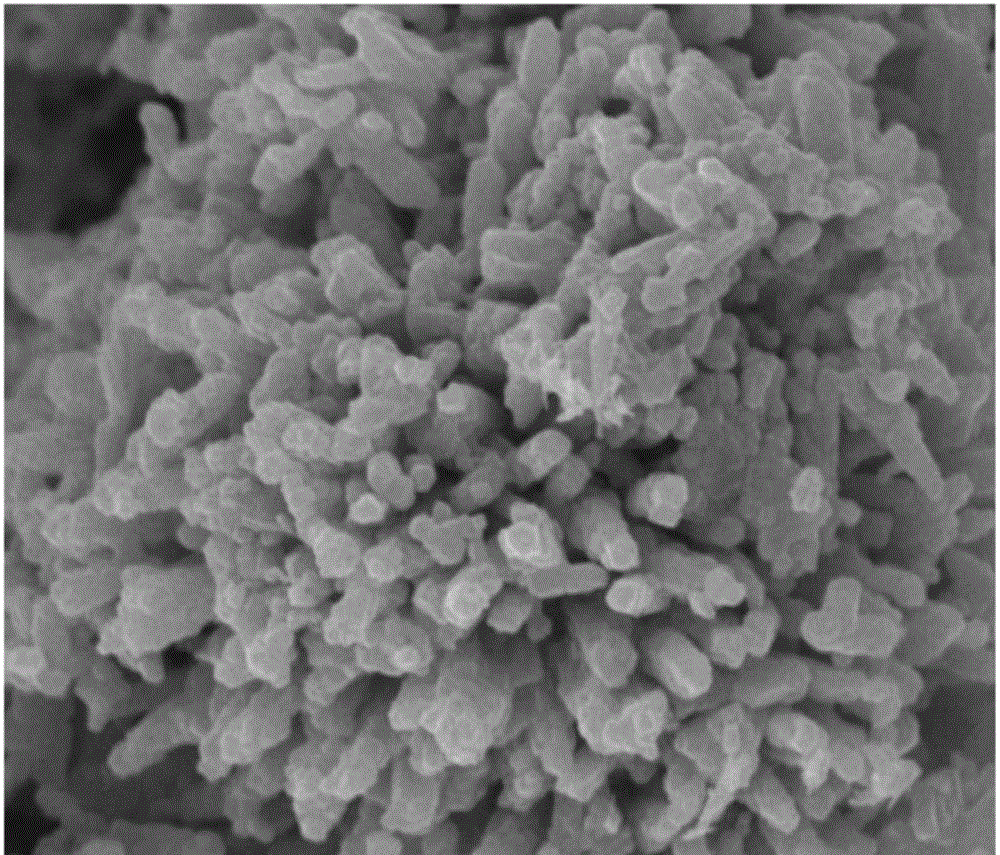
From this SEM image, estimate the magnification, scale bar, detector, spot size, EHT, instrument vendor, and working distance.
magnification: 10 K X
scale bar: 2000 nm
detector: ETD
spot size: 3.5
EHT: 15 kV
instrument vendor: FEI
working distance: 10.6 mm